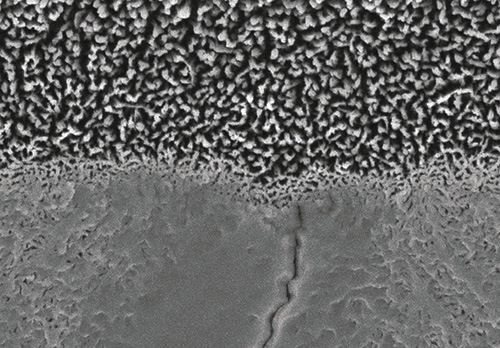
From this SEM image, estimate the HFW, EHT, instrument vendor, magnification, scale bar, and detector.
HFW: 6 µm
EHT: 5 kV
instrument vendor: other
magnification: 20 K X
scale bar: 1000 nm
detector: A+B